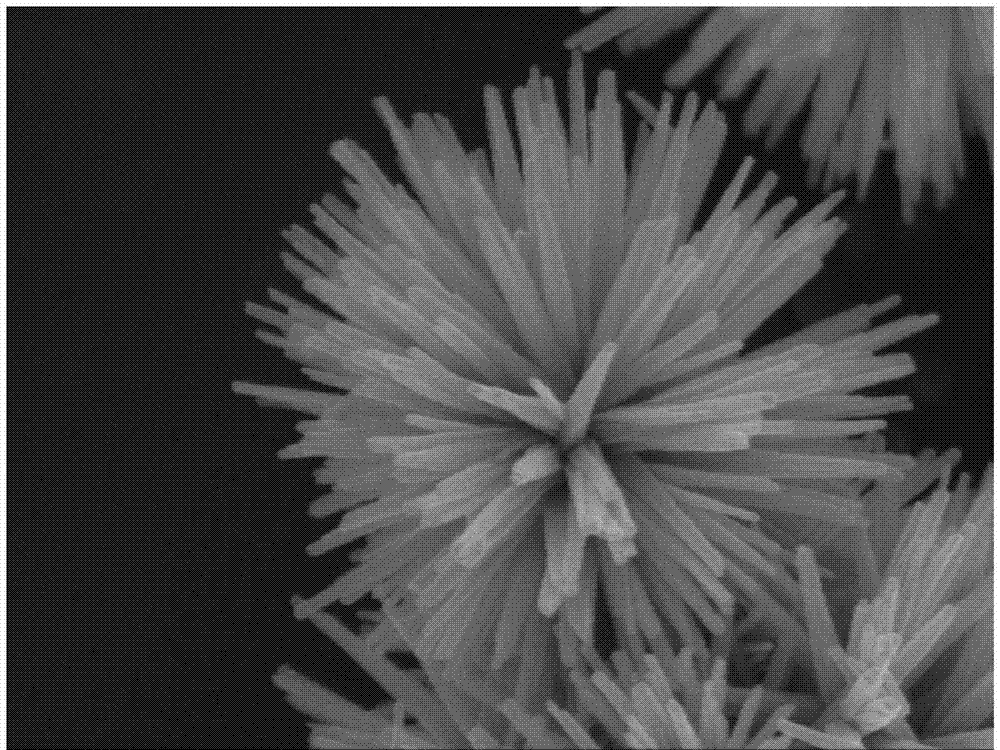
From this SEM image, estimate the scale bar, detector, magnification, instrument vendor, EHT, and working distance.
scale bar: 100 nm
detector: SEI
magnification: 50 K X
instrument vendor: JEOL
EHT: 5 kV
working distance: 8.5 mm